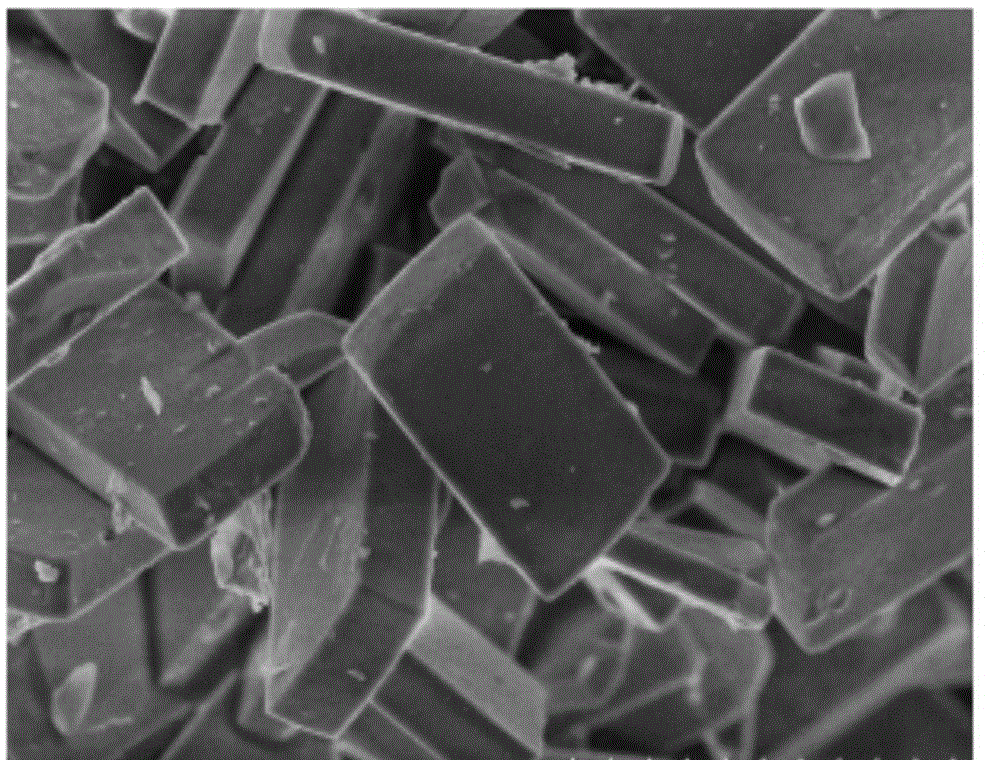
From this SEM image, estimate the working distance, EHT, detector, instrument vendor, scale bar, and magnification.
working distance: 6.6 mm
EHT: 3 kV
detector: SE(M)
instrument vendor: Hitachi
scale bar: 5000 nm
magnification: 10 K X